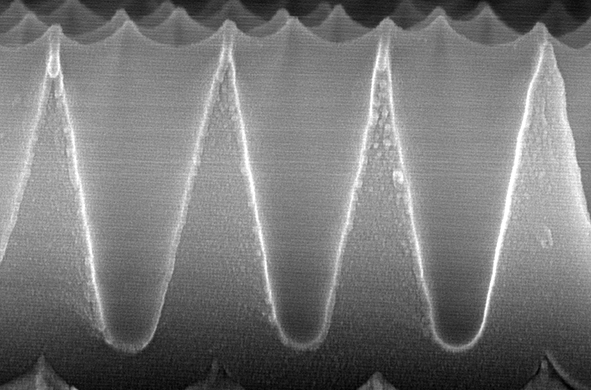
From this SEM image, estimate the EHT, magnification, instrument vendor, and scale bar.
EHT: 10 kV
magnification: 70 K X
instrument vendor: Hitachi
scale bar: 300 nm